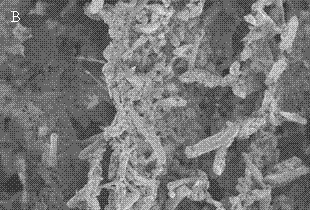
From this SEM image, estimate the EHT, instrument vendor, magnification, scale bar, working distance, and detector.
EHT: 3 kV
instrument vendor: Hitachi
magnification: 0.3 K X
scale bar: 100000 nm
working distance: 10.2 mm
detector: SE(M)